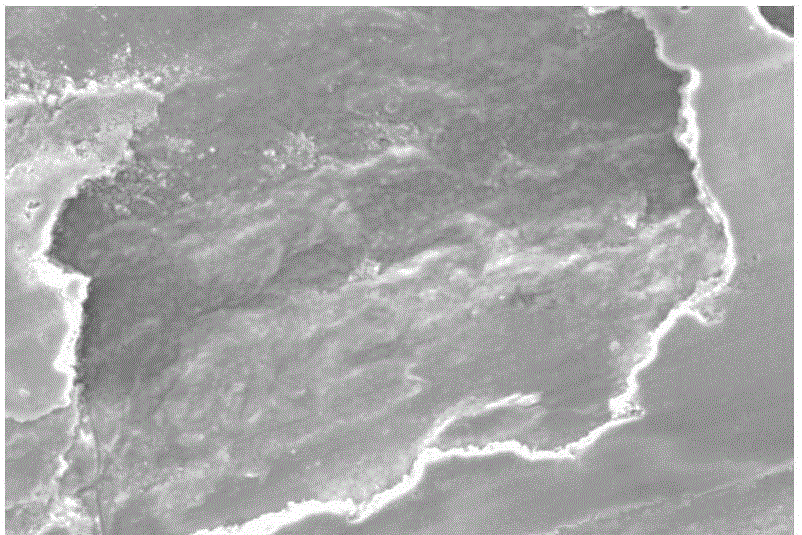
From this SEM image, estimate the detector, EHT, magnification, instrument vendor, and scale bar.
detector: SEI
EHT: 25 kV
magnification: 0.5 K X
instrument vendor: JEOL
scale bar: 50000 nm